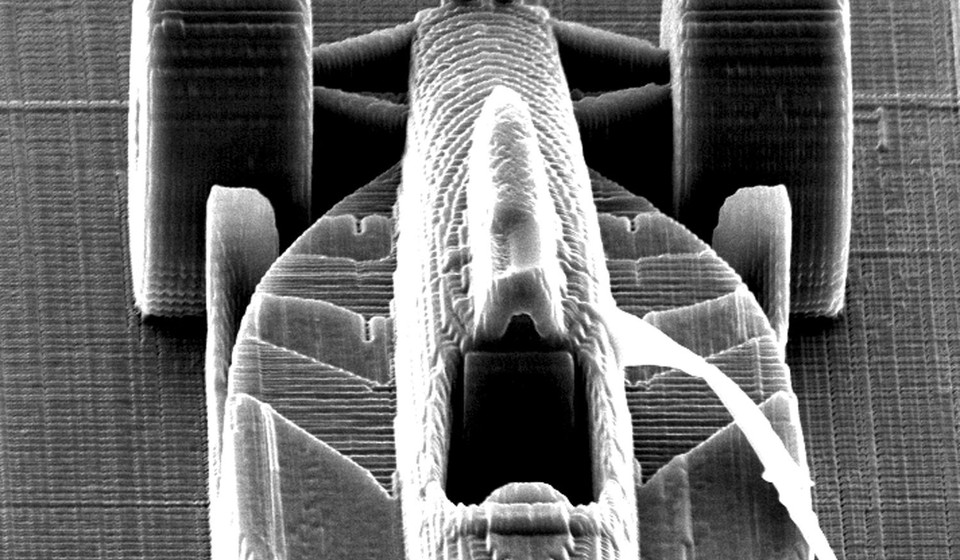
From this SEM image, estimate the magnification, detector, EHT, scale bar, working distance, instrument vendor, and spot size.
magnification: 0.729 K X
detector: SE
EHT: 20 kV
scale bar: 50000 nm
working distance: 27.2 mm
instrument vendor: FEI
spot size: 4